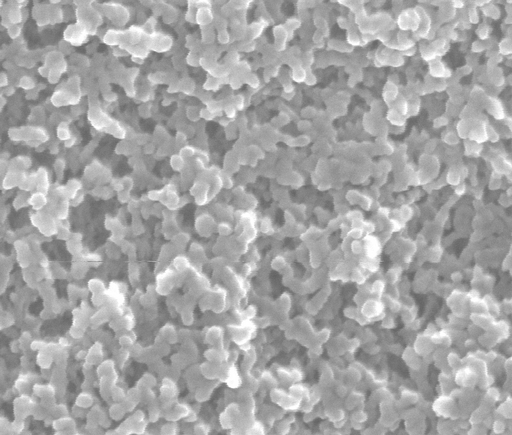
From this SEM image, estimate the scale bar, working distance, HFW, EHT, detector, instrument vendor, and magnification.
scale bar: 300 nm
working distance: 10 mm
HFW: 1.35 µm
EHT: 20 kV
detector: LFD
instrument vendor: FEI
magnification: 100 K X